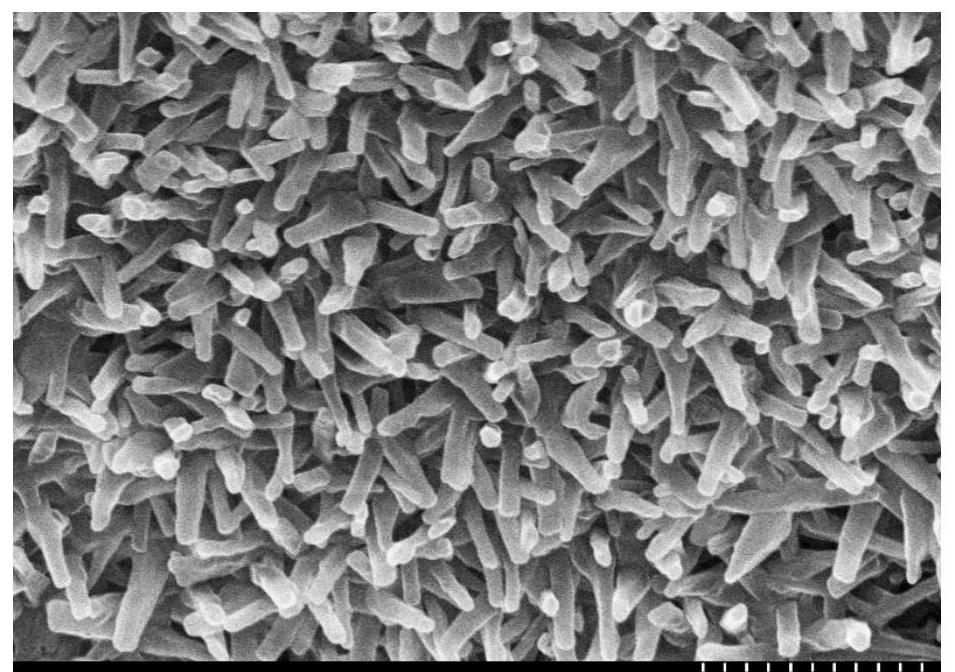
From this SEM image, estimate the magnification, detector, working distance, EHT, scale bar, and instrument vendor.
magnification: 30 K X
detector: SE(M)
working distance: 8.6 mm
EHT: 15 kV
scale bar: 1000 nm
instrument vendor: Hitachi